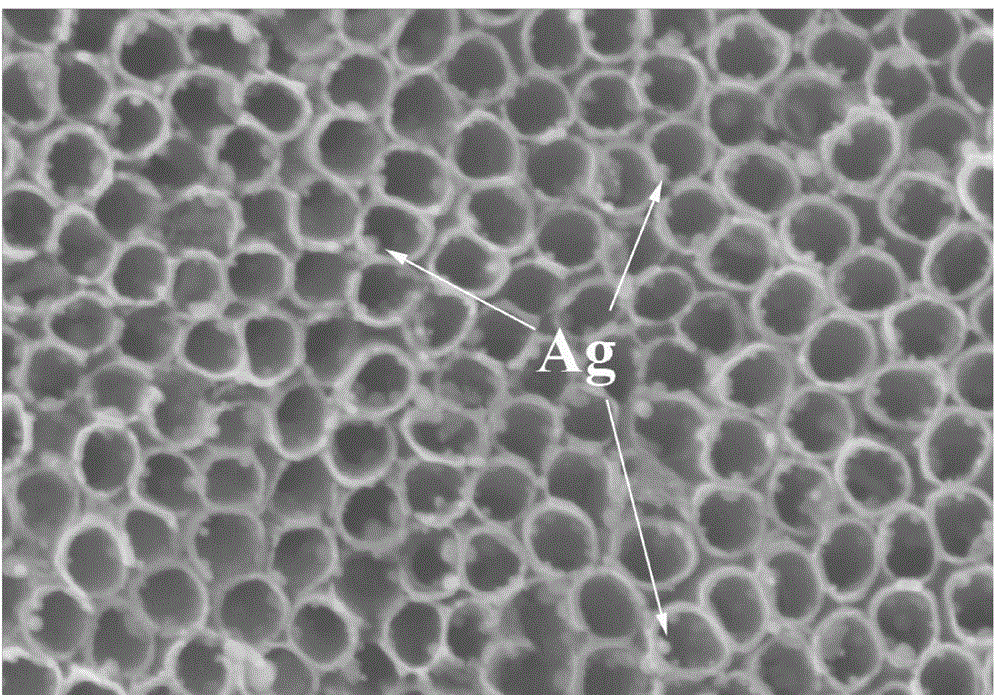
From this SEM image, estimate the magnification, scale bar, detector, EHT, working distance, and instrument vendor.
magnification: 100 K X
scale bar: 500 nm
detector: SE(M)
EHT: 10 kV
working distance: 8.9 mm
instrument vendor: Hitachi